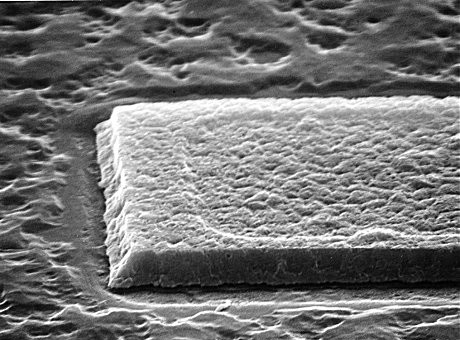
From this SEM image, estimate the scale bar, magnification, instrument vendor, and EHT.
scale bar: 10000 nm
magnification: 5 K X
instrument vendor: JEOL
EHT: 30 kV